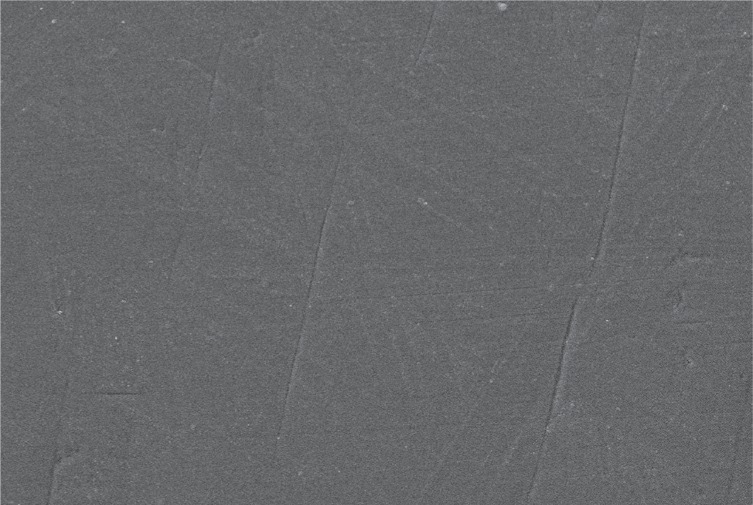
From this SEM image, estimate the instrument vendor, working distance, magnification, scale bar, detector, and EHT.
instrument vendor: JEOL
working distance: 10 mm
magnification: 1 K X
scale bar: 10000 nm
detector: SEI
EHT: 20 kV